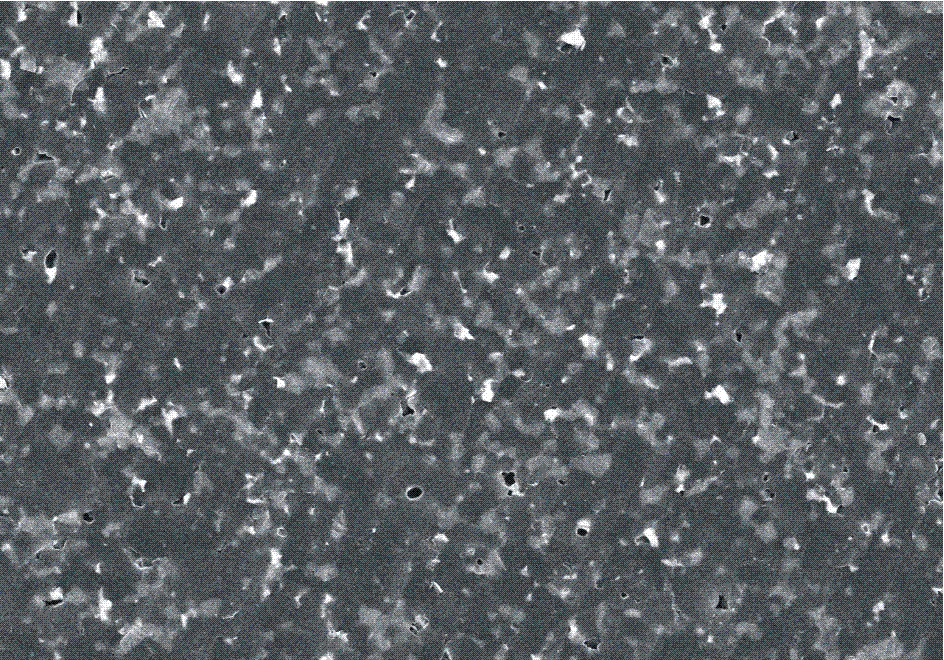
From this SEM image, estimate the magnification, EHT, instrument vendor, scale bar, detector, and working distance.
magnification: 2 K X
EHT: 15 kV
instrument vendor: Hitachi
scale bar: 20000 nm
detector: SE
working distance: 11.9 mm